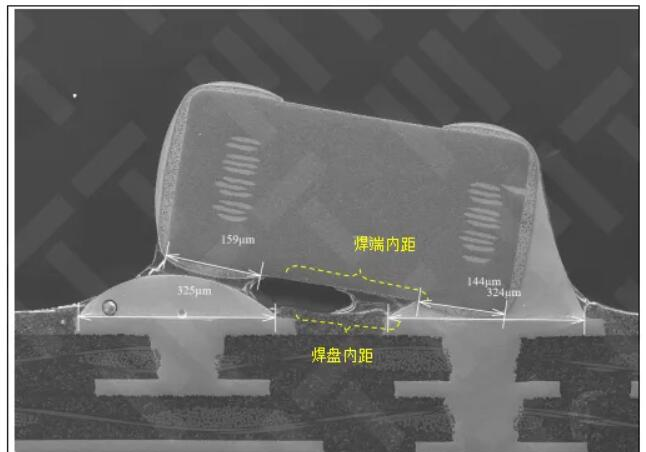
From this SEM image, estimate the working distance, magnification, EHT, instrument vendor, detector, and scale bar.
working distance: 10.4 mm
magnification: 0.12 K X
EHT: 15 kV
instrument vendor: Hitachi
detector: LM(UL)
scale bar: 400000 nm